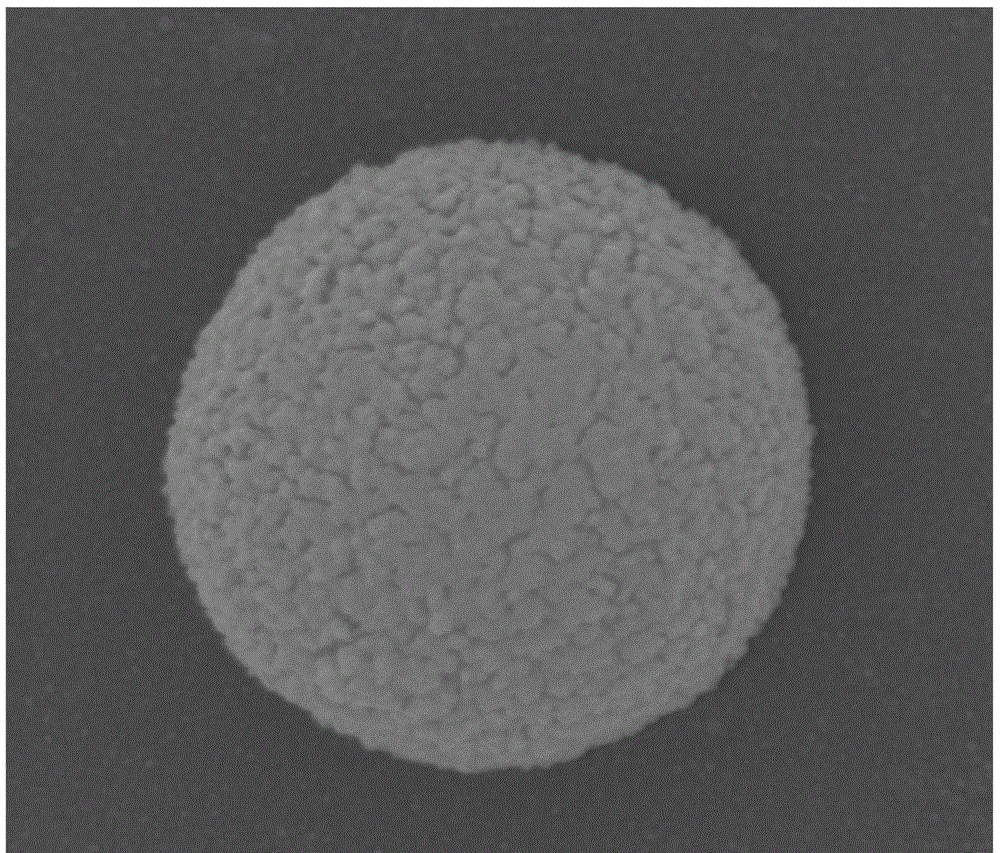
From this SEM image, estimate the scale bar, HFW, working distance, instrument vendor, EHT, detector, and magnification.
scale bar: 500 nm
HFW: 2.4 µm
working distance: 9.9 mm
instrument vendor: FEI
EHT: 20 kV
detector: ETD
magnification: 100 K X